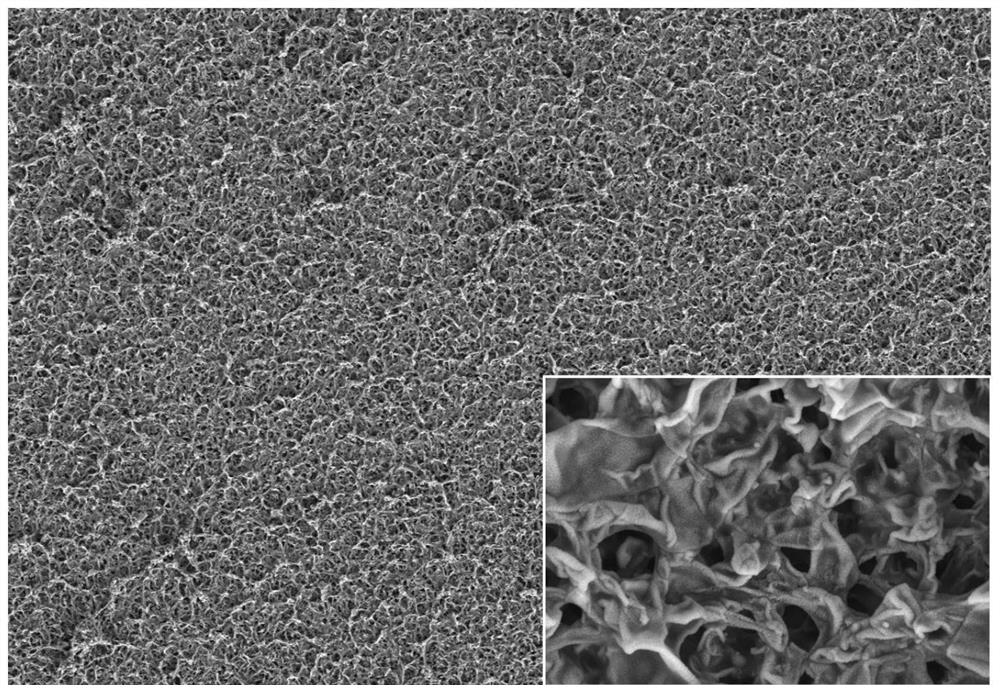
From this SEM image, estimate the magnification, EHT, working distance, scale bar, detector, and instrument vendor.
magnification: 1.7 K X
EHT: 3 kV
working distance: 5.4 mm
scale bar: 5000 nm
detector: SE2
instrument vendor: Zeiss